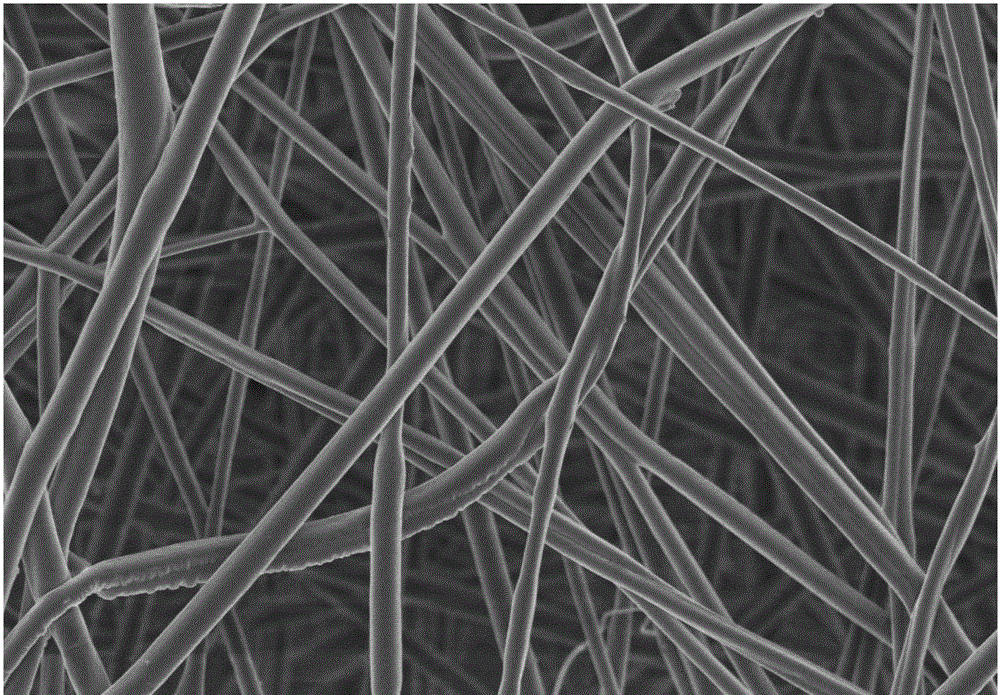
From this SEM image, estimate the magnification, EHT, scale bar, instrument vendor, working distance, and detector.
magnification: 10 K X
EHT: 2 kV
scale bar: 5000 nm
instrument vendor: Hitachi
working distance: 7.5 mm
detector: SE(U)